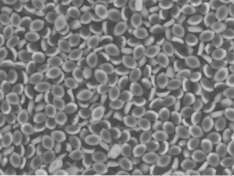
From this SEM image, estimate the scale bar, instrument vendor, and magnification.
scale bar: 50000 nm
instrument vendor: Hitachi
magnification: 1.8 K X